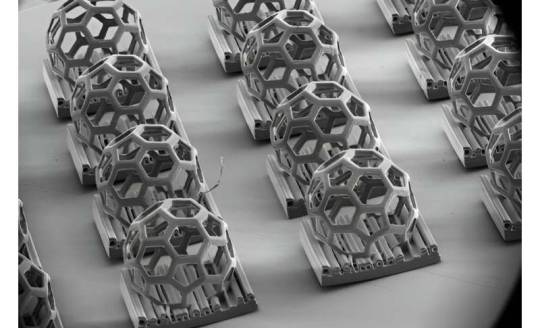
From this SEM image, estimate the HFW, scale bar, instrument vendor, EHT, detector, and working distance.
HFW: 3190 µm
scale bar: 1000000 nm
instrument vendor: Thermo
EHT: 2 kV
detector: ETD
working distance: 15.8 mm